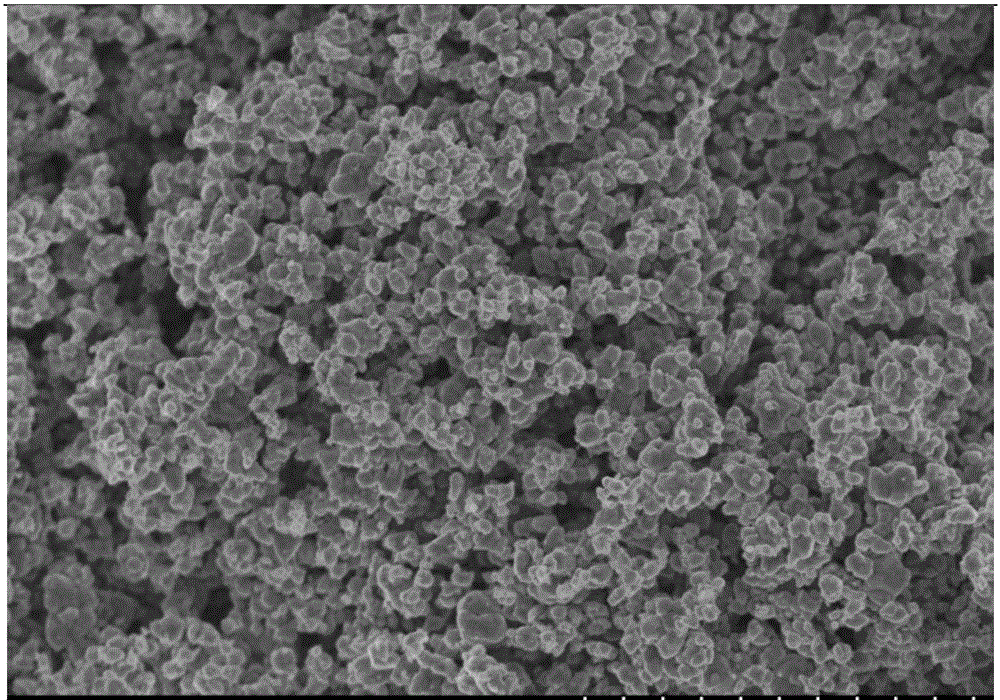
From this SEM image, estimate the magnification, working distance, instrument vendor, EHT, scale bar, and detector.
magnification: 10 K X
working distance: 8 mm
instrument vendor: Hitachi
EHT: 3 kV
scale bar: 5000 nm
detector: SE(M)